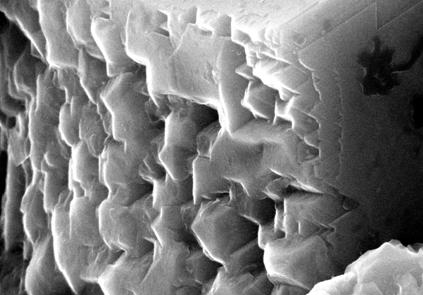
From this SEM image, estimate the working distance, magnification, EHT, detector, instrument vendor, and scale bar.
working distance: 4.6 mm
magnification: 75.5 K X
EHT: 20 kV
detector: InLens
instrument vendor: Zeiss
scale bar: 1000 nm